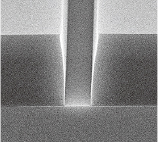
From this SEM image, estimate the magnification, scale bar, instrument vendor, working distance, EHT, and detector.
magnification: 6 K X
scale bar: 5000 nm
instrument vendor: Hitachi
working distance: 5.4 mm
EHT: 5 kV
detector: SE(U)